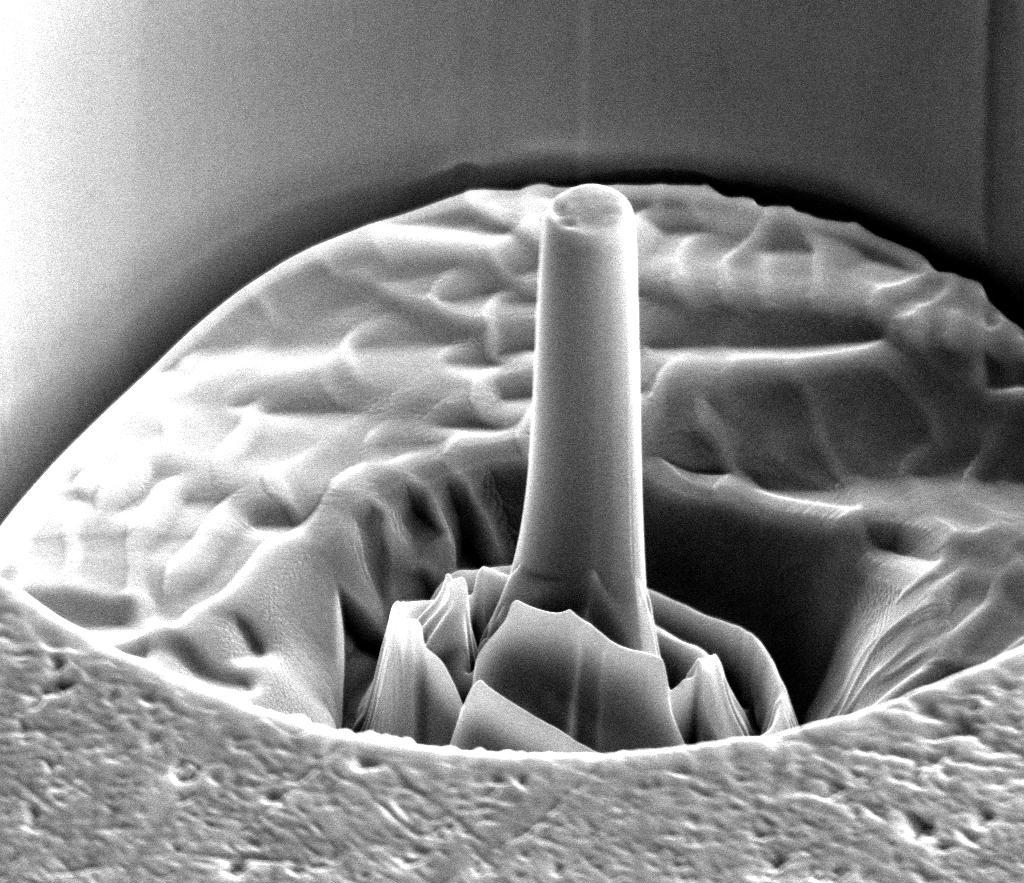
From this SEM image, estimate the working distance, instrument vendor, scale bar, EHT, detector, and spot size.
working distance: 9.9 mm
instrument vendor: FEI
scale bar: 4000 nm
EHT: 10 kV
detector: ETD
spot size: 4